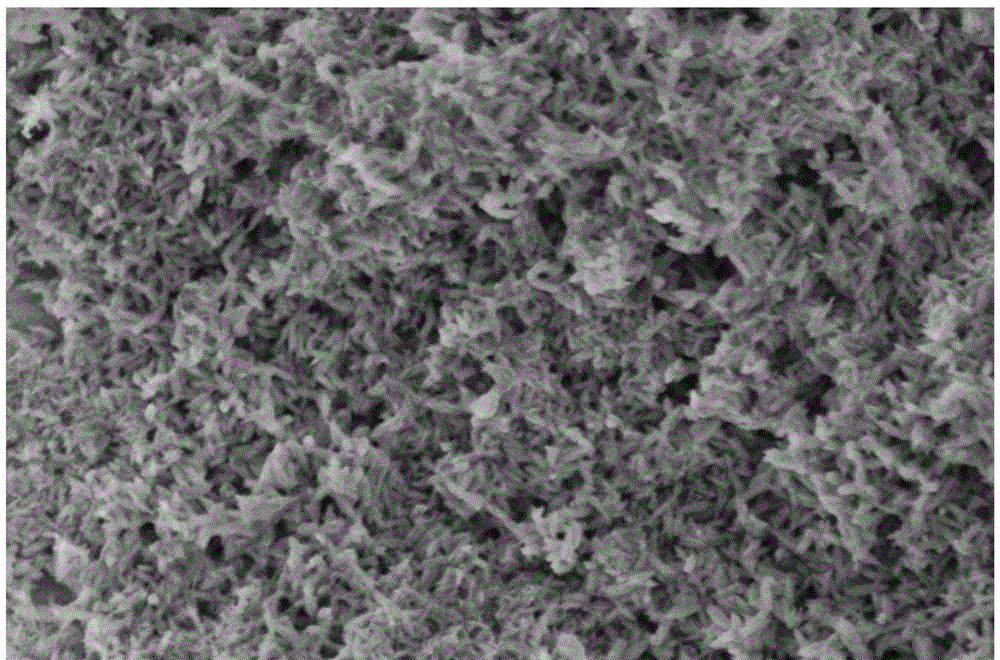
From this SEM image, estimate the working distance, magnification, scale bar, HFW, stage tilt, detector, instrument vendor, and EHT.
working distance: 3 mm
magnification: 30 K X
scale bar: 1000 nm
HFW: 4.23 µm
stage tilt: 0°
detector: TLD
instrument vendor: FEI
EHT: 2 kV